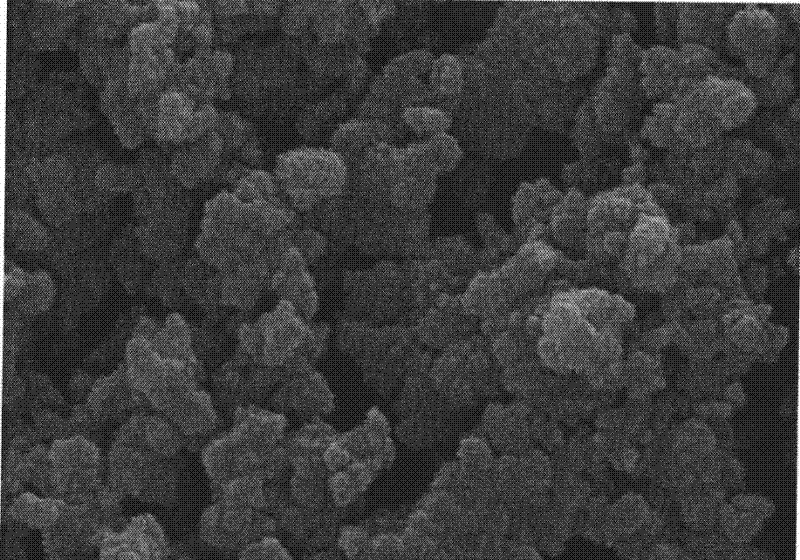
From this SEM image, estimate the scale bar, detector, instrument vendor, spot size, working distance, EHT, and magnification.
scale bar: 500 nm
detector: SE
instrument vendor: FEI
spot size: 3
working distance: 5 mm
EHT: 10 kV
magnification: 40 K X